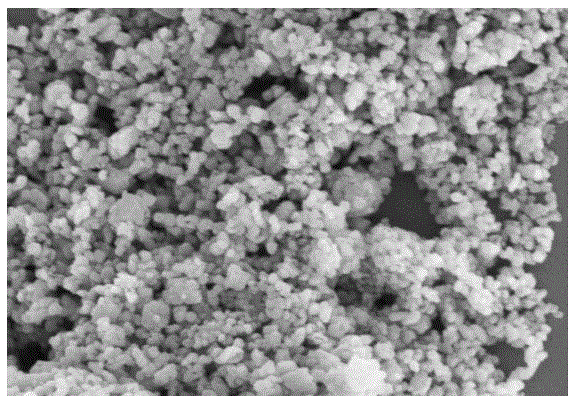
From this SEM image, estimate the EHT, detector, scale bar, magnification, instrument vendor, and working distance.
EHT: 5 kV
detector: SE(M)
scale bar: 1000 nm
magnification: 30 K X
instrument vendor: Hitachi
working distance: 9.8 mm